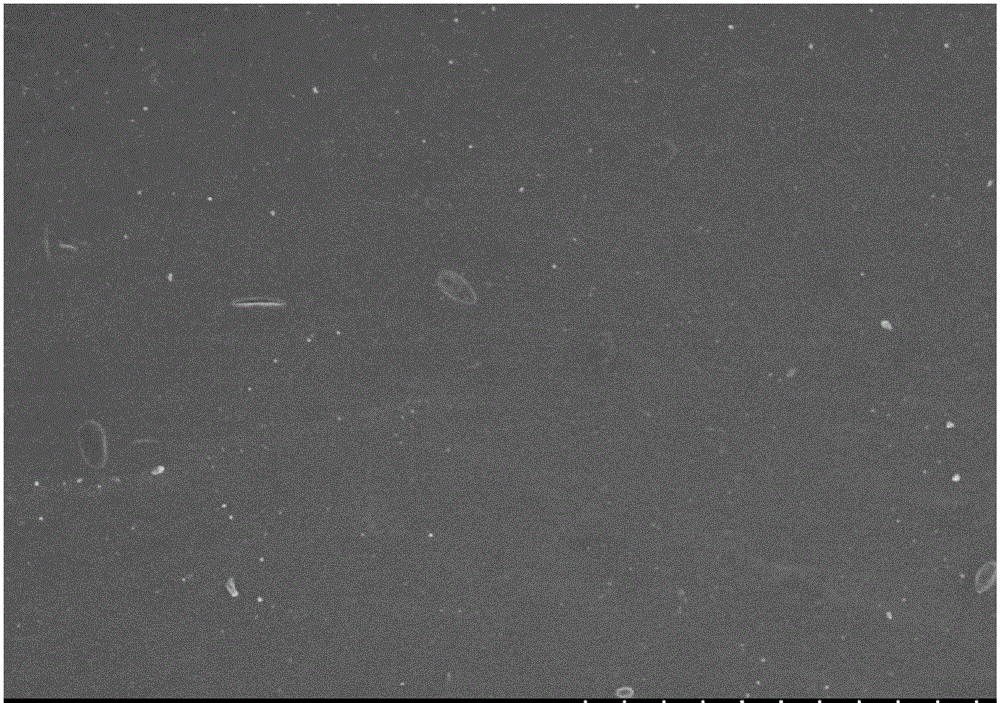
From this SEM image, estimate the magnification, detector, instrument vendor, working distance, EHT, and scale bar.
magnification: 1 K X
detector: SE(M,LA0)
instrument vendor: Hitachi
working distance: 8.8 mm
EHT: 5 kV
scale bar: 50000 nm